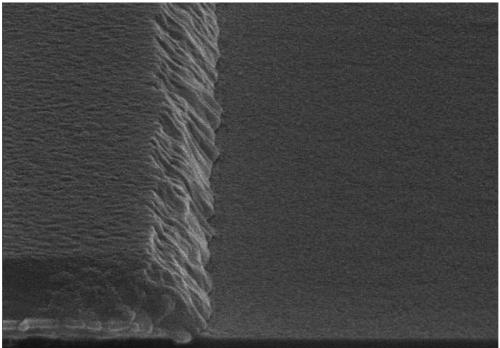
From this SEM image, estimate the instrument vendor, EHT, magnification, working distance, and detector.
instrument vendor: Hitachi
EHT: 15 kV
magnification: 30 K X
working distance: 13.5 mm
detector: SE(U)